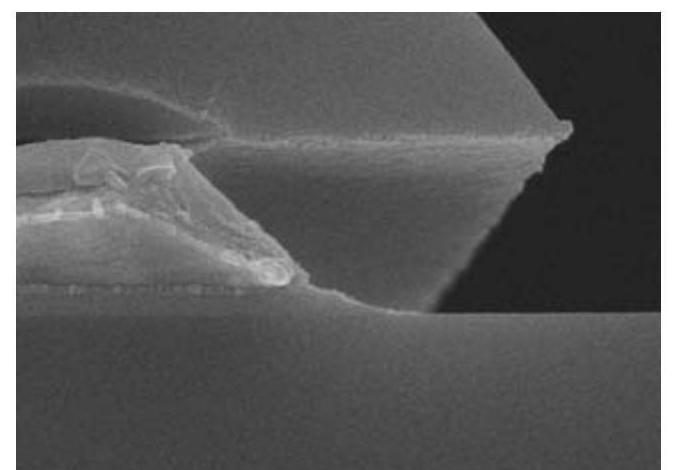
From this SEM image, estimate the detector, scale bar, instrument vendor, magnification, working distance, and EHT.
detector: SE(U)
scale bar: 1000 nm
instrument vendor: Hitachi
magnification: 50 K X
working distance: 12.1 mm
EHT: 10 kV